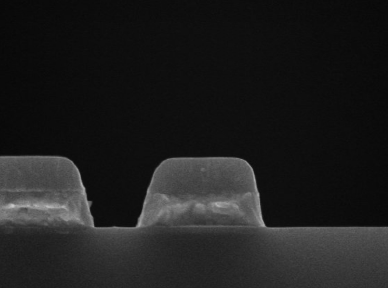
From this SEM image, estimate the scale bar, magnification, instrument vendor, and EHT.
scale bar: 600 nm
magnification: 50 K X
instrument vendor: Hitachi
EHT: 10 kV